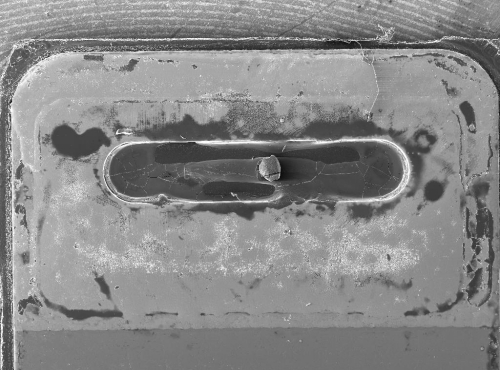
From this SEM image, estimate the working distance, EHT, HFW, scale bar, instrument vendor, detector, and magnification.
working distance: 80.39 mm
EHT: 20 kV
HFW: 8110 µm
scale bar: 2000000 nm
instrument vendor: Tescan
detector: SE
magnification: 0.034 K X